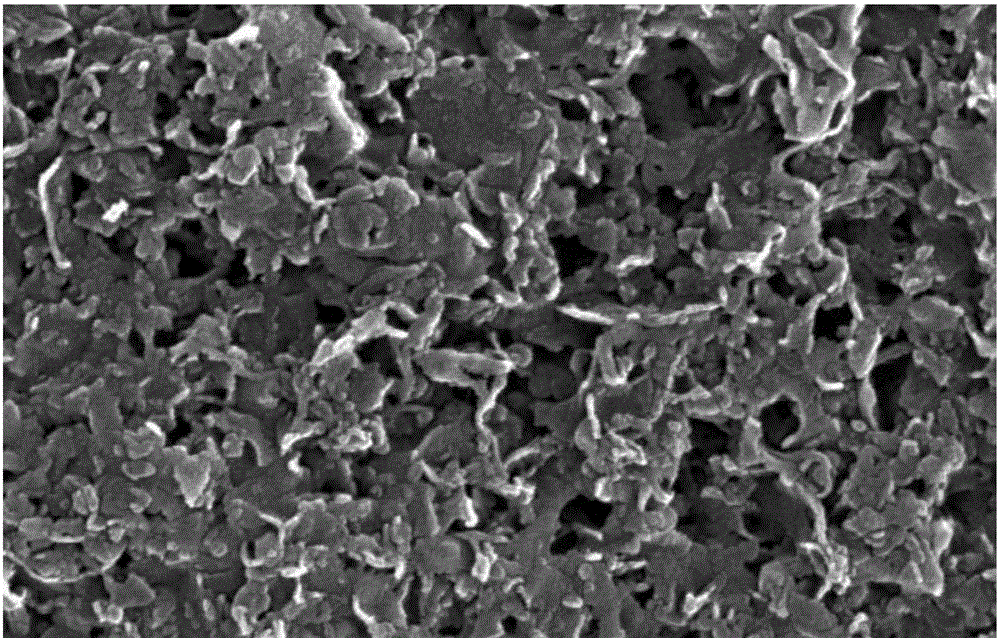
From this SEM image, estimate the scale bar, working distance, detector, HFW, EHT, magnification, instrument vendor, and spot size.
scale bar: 1000 nm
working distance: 8 mm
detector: ETD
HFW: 4.14 µm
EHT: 20 kV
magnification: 100 K X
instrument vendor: FEI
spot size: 3